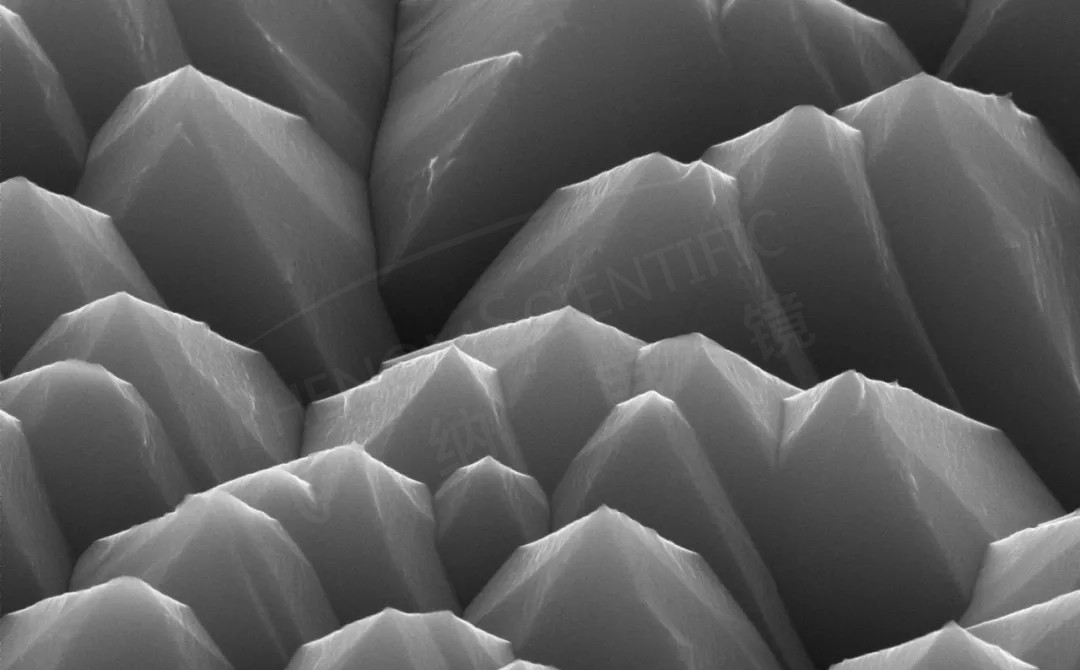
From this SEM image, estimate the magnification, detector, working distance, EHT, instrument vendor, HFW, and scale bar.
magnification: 20 K X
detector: SED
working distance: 7.389 mm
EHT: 15 kV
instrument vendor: Thermo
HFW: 6.35 µm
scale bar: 2000 nm